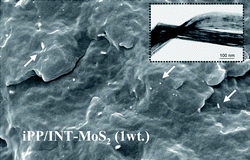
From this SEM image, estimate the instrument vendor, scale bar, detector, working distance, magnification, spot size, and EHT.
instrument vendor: FEI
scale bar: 5000 nm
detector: SE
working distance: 7.3 mm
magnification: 5 K X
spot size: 2.5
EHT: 25 kV